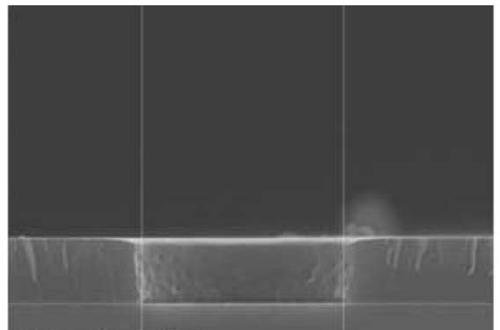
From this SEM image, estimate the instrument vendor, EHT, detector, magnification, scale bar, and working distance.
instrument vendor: JEOL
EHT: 15 kV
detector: SEI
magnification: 4 K X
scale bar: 1000 nm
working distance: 7.8 mm